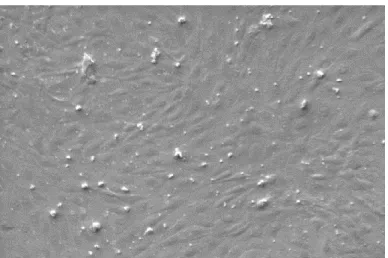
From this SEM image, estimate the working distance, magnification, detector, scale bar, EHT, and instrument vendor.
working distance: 14.5 mm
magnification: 0.558 K X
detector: SE1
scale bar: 20000 nm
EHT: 4 kV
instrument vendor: Zeiss